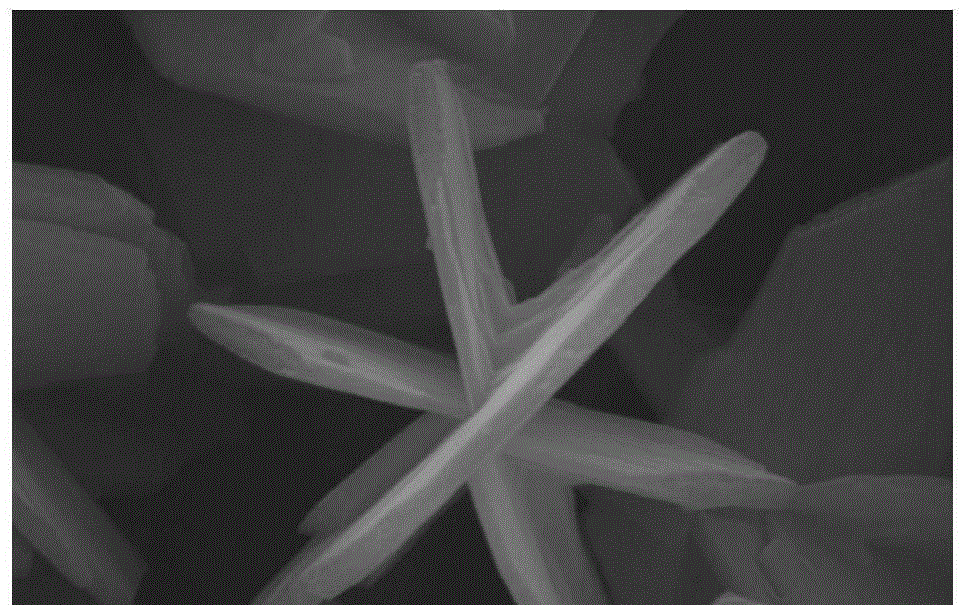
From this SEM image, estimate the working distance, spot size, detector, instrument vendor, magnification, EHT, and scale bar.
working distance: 6 mm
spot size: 3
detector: SE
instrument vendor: FEI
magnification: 31.621 K X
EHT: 20 kV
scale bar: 1000 nm